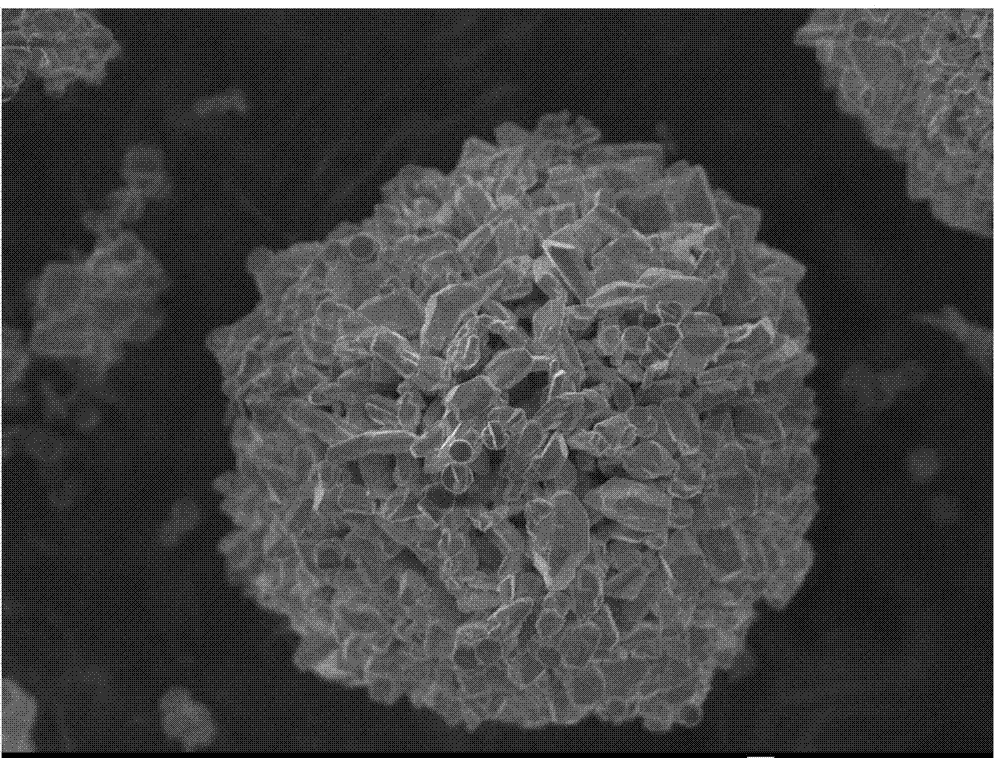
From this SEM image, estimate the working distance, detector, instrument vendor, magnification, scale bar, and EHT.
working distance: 7.9 mm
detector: SEI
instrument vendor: JEOL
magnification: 3.3 K X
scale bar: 1000 nm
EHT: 5 kV